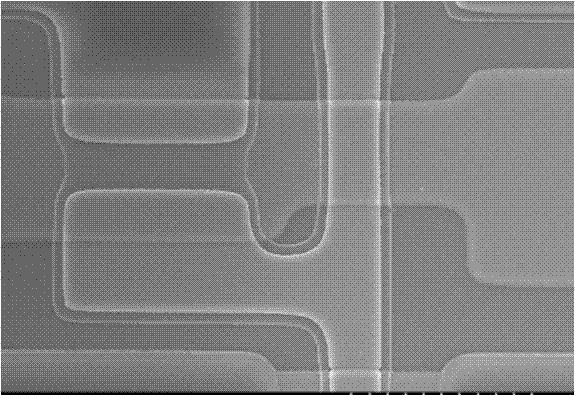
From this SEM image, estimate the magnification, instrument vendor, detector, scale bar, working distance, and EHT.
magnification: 2 K X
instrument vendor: Hitachi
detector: SE(U)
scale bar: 20000 nm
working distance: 11.1 mm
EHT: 15 kV